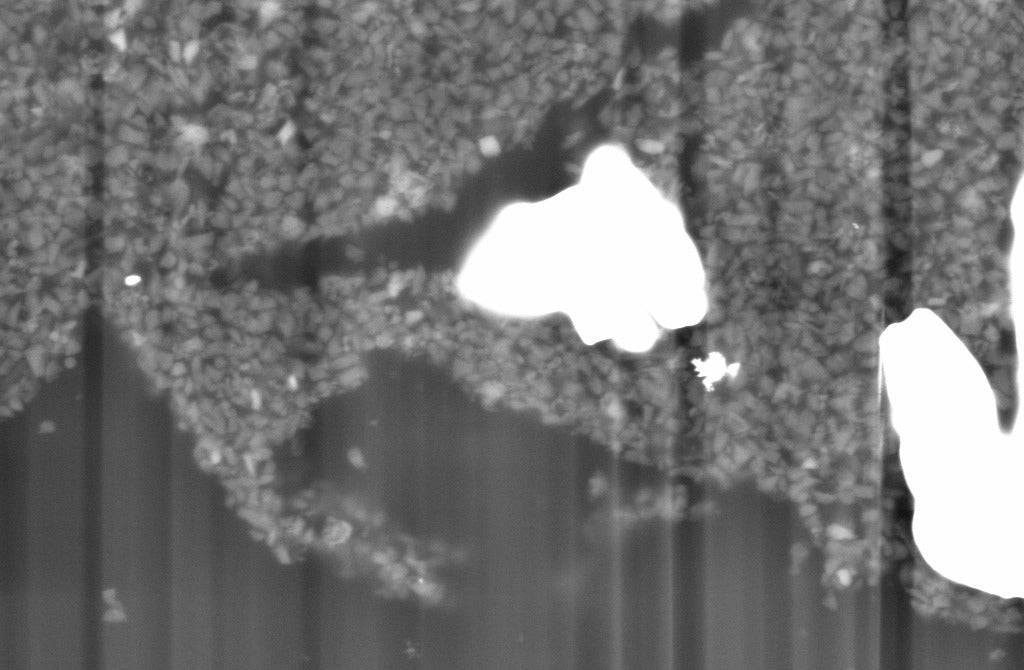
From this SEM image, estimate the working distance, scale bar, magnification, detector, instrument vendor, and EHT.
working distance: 10.1 mm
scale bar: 1000 nm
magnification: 10 K X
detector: BSD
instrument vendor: Zeiss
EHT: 10 kV